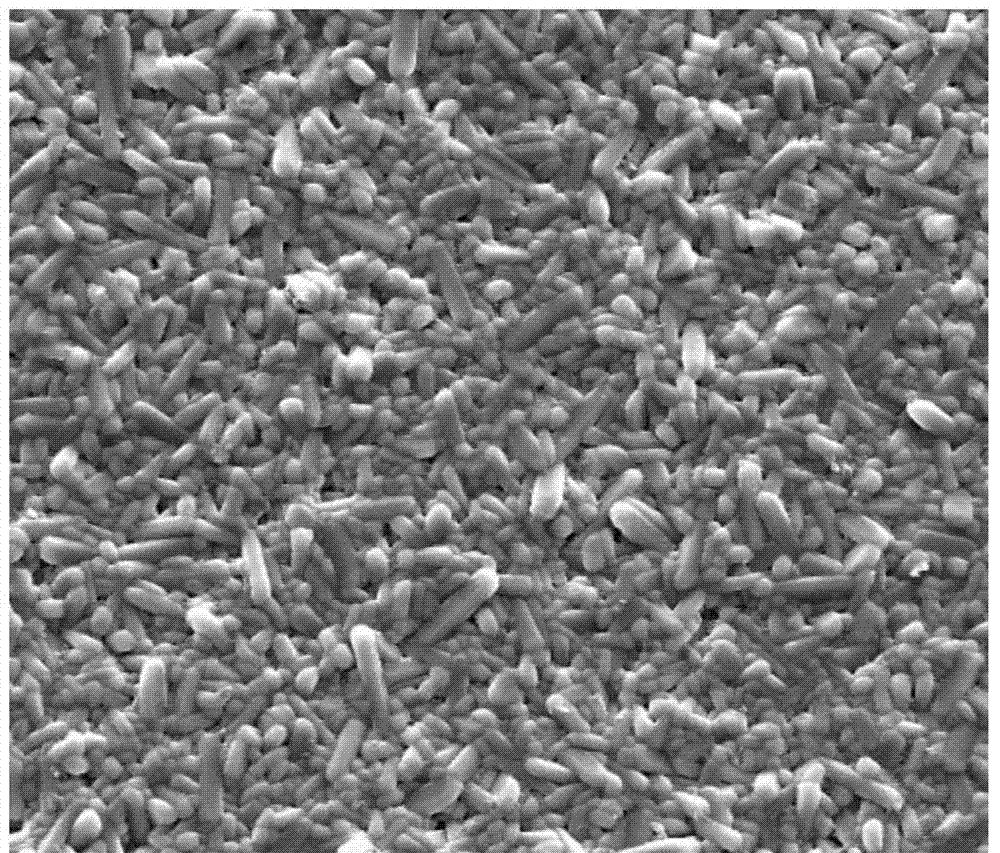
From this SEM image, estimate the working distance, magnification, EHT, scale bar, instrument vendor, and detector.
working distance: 9 mm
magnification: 5 K X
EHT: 20 kV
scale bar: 20000 nm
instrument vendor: FEI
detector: SE